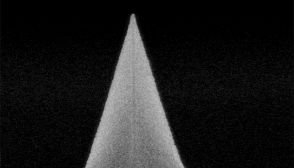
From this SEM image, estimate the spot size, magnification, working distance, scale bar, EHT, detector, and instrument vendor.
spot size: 3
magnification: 6.5 K X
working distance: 4 mm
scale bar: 6000 nm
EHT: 24 kV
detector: SE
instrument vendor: FEI